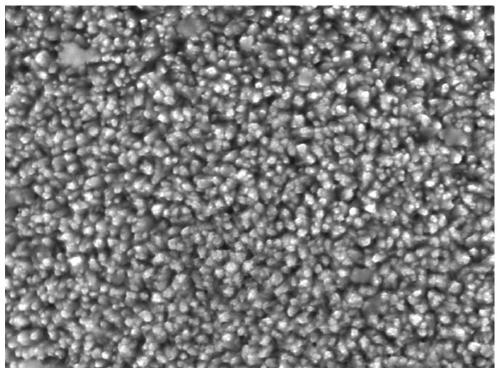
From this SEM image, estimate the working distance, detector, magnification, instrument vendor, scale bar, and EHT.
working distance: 20.7 mm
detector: LED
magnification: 10 K X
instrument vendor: JEOL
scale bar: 1000 nm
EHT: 15 kV